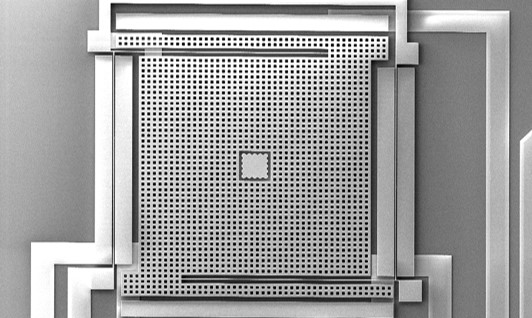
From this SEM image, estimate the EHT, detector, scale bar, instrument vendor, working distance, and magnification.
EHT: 10 kV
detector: SE2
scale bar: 100000 nm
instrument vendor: Zeiss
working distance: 18 mm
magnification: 0.4 K X